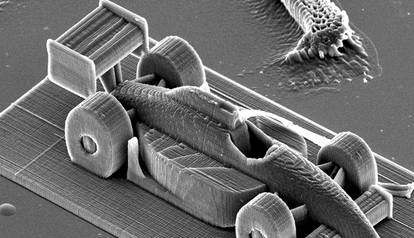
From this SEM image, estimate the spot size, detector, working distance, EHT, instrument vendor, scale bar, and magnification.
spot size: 4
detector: SE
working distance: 27.2 mm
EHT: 20 kV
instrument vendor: FEI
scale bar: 100000 nm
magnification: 0.364 K X